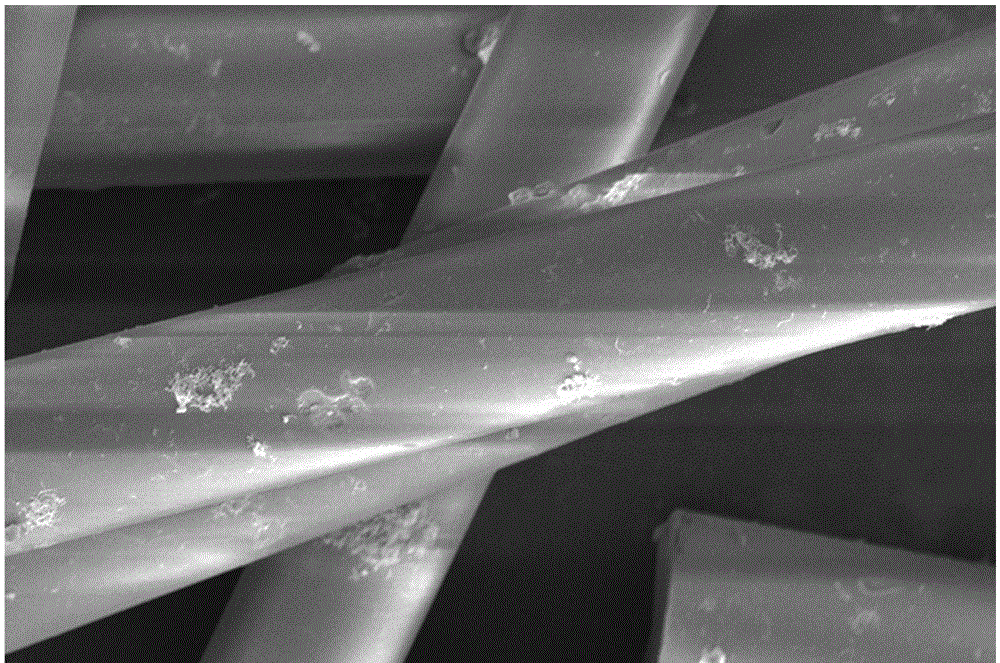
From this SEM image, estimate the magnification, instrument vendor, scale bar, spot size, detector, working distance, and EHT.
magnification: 6 K X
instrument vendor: FEI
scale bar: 30000 nm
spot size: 3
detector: ETD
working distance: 5.1 mm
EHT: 5 kV